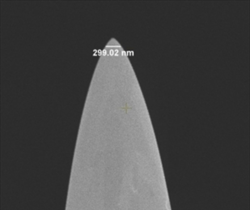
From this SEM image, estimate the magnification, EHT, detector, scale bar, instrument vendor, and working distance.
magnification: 60 K X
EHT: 3 kV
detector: TLD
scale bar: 2000 nm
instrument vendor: FEI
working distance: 5.2 mm